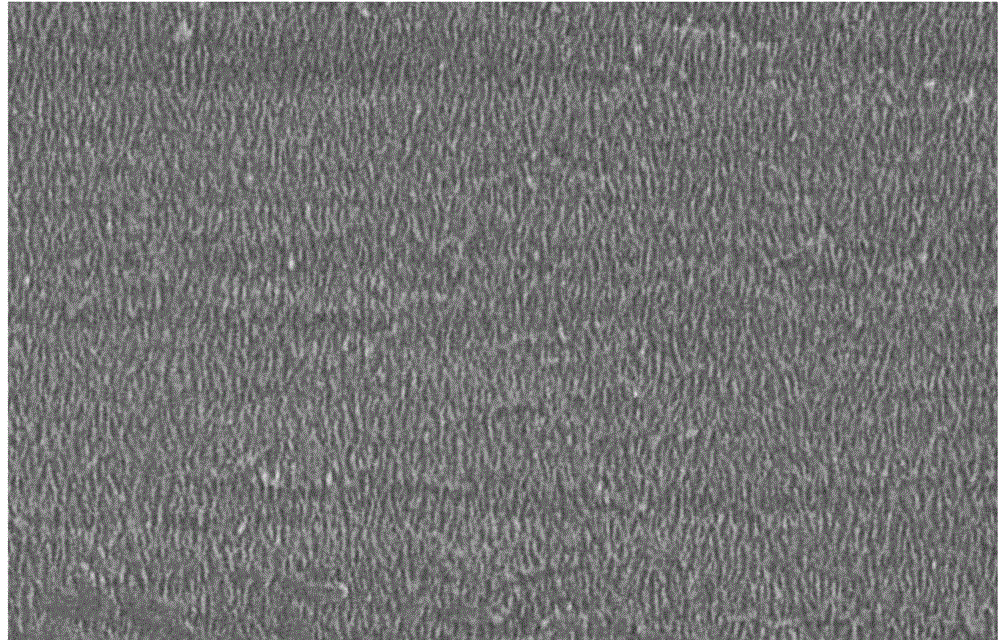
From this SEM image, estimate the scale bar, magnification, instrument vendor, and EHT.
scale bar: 1000 nm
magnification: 10 K X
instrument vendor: JEOL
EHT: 20 kV